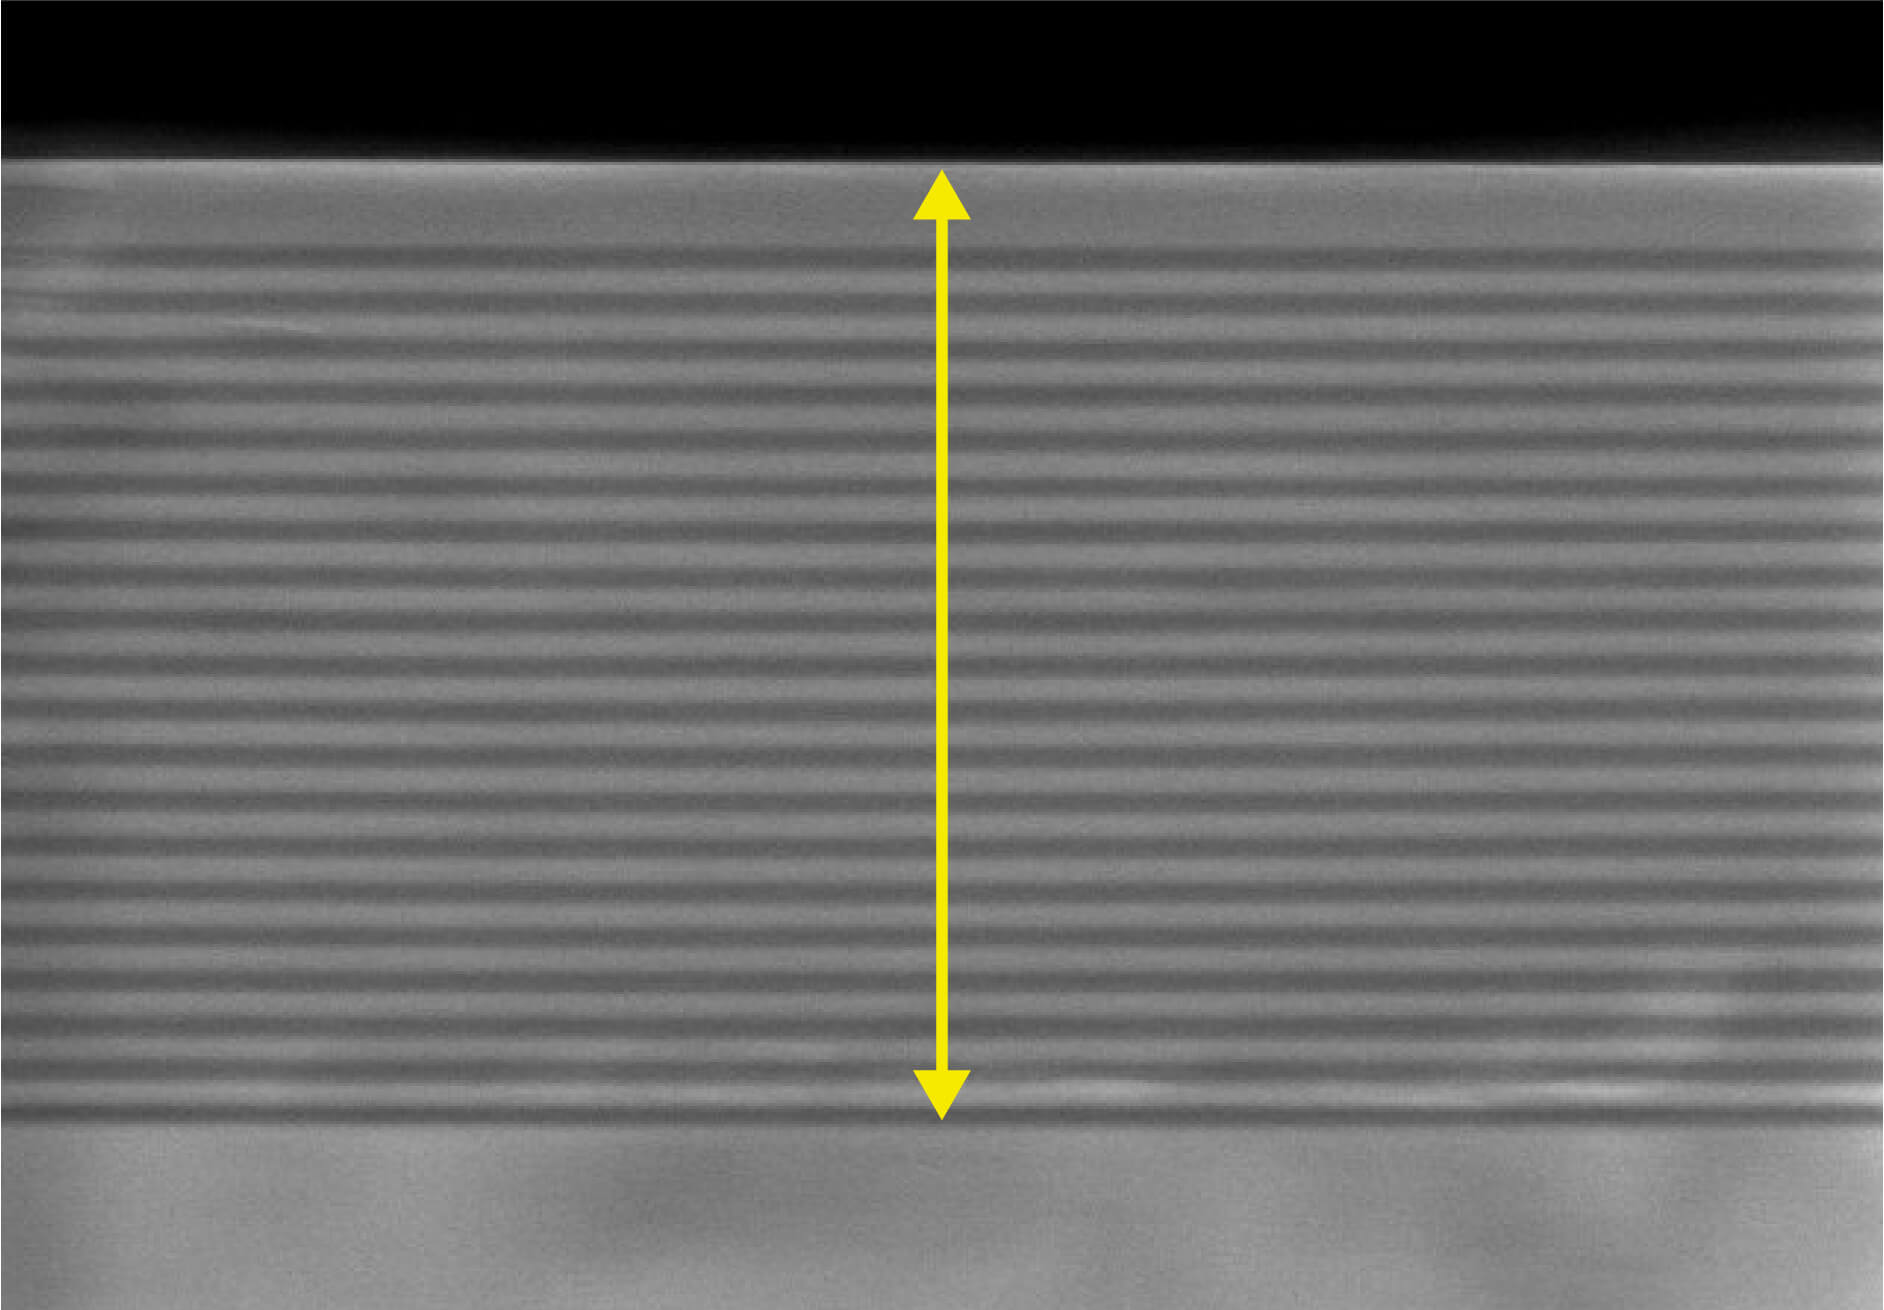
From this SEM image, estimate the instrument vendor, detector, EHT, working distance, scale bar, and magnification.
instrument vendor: Hitachi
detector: SE(U)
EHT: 3 kV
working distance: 9 mm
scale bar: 1000 nm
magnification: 45 K X